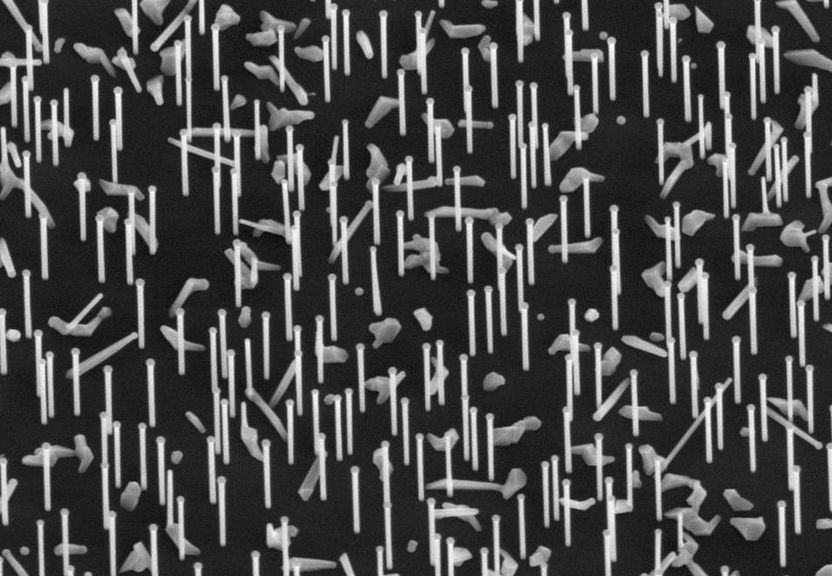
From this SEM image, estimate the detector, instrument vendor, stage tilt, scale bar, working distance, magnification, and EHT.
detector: T2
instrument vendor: FEI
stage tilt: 30°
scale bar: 2000 nm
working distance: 6.9 mm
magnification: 30 K X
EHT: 5 kV